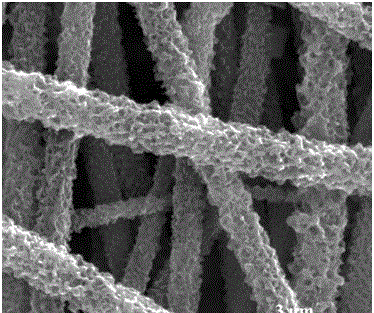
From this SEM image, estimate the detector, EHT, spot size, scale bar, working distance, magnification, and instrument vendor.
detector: ETD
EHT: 30 kV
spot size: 3.5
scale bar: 3000 nm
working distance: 9.4 mm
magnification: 40 K X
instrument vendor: FEI